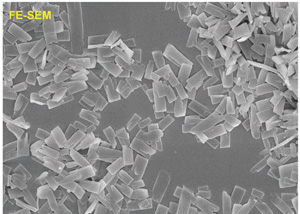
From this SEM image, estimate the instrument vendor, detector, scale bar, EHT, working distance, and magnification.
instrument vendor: JEOL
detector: SEI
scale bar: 1000 nm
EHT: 5 kV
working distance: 8.5 mm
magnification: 12 K X